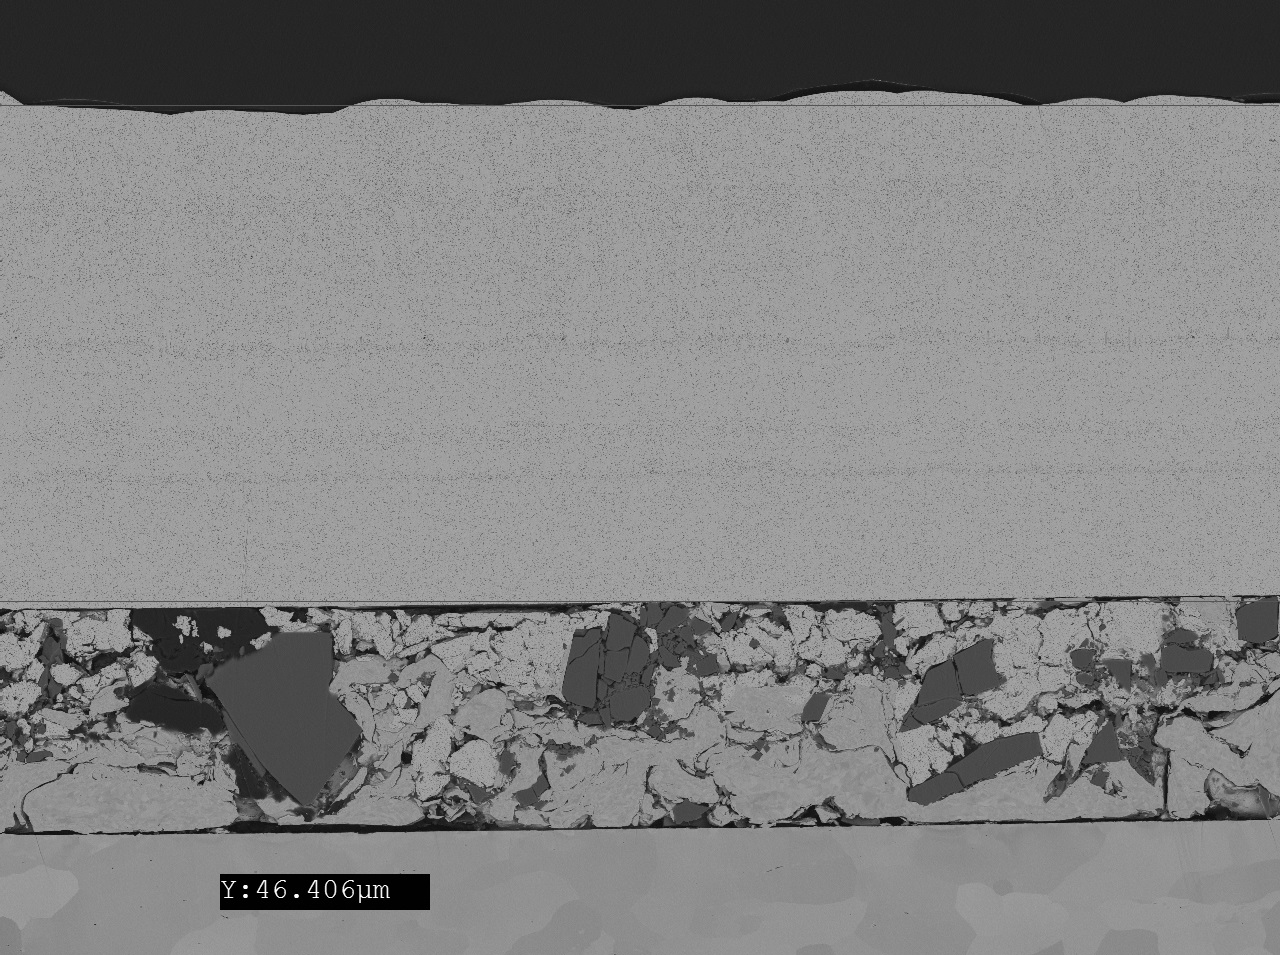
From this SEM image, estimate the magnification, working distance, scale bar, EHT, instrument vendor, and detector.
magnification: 1 K X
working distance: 9.7 mm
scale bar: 10000 nm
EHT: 5 kV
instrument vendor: JEOL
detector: BED-C